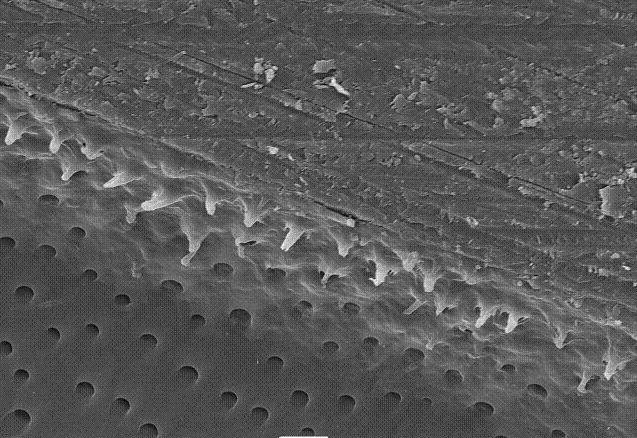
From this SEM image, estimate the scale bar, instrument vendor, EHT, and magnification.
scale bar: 10000 nm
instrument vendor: JEOL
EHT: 15 kV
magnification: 1 K X